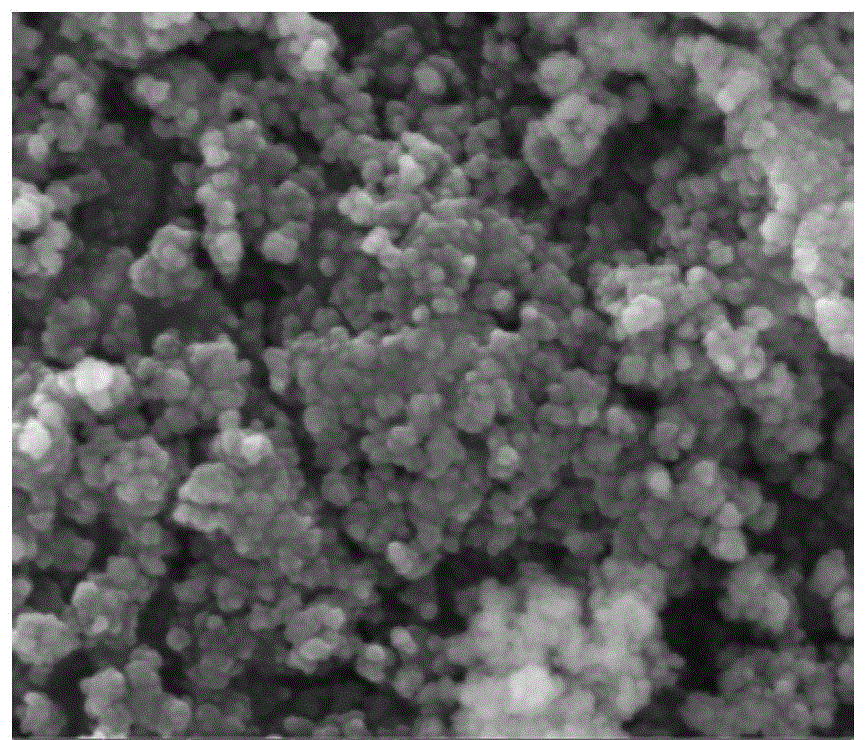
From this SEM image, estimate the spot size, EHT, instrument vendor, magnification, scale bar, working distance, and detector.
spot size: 3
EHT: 10 kV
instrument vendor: FEI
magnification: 100 K X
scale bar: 1000 nm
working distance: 8.1 mm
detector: ETD